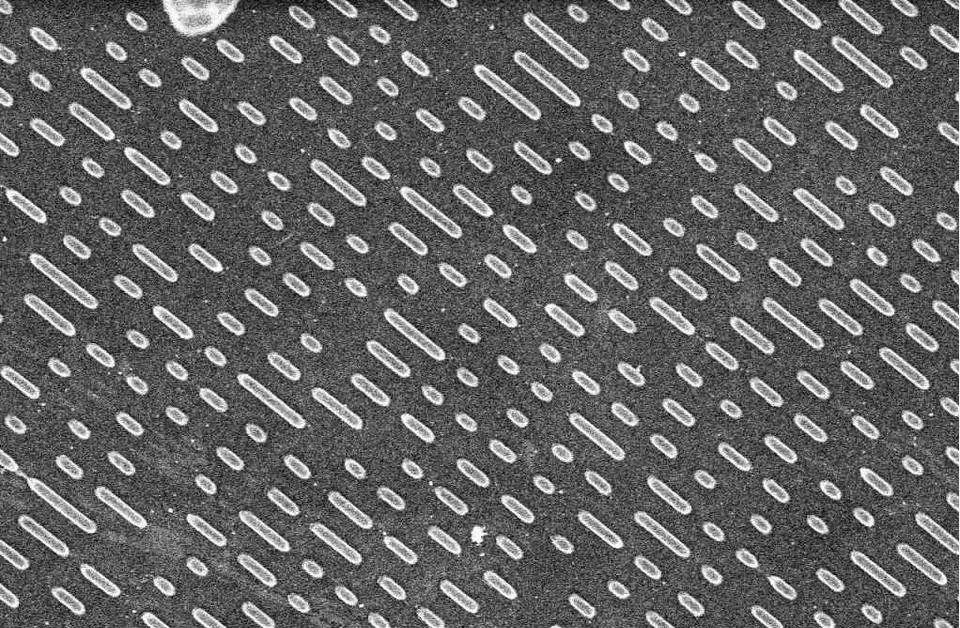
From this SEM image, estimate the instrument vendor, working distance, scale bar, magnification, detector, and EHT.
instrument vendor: Hitachi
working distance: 5 mm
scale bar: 10000 nm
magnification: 3 K X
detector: SE(M)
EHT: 5 kV